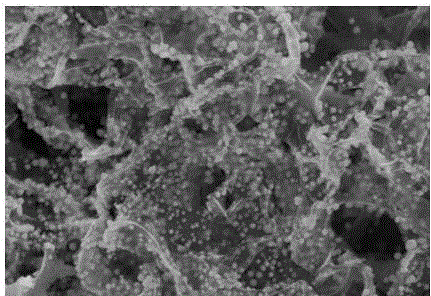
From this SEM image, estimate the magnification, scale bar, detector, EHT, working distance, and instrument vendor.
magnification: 50 K X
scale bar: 1000 nm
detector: SE(M)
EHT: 5 kV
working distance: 8.2 mm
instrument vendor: Hitachi